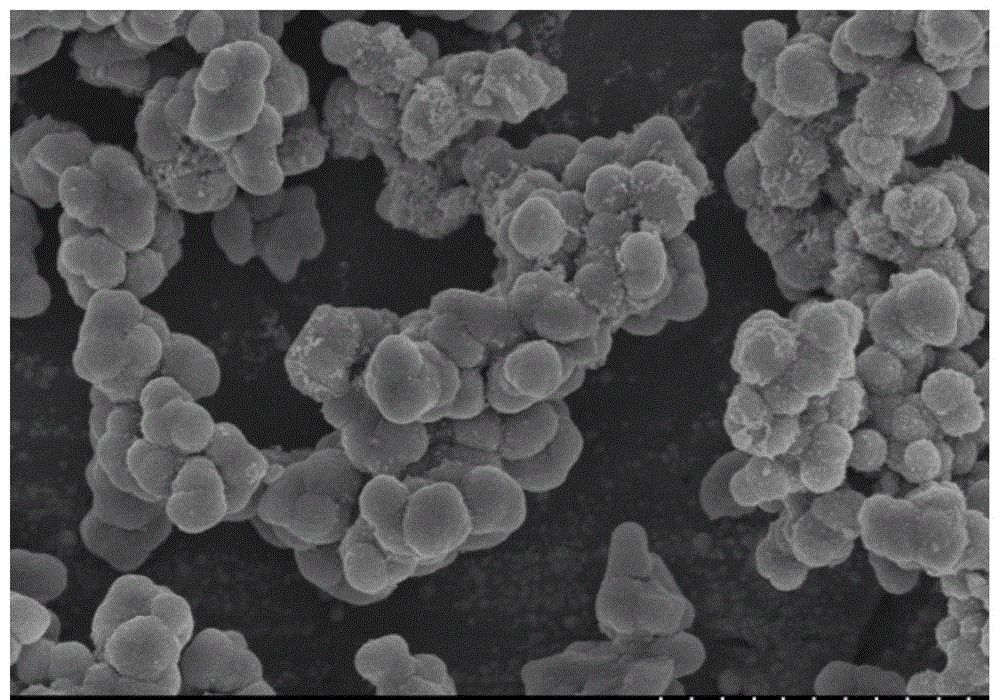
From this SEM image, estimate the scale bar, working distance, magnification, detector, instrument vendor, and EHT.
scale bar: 2000 nm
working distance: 9.3 mm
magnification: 20 K X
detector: SE(M)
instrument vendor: Hitachi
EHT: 5 kV